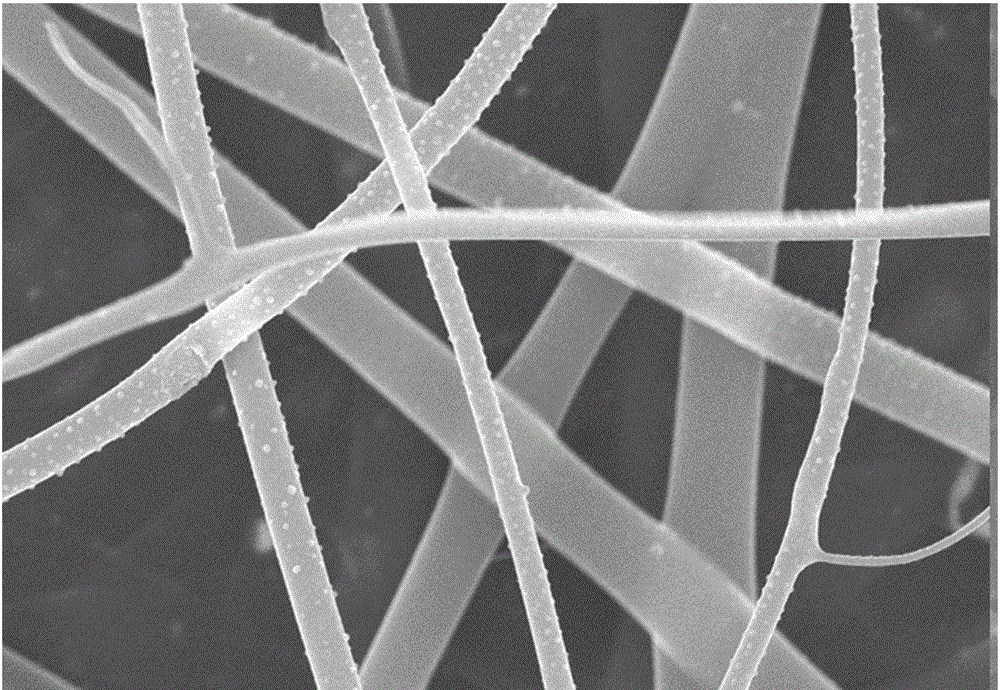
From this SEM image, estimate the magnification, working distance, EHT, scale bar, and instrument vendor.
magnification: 10 K X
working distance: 12.5 mm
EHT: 20 kV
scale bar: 5000 nm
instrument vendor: Hitachi